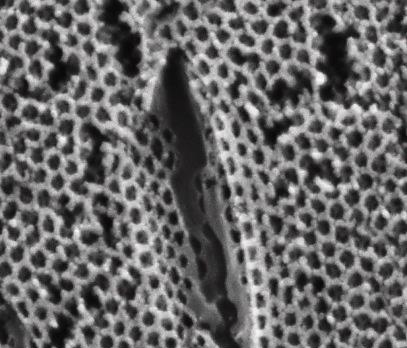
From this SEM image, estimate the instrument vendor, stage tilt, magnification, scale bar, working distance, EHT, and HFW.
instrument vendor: FEI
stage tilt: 0°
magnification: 50 K X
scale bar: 2000 nm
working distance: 10.7 mm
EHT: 25 kV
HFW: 5.41 µm